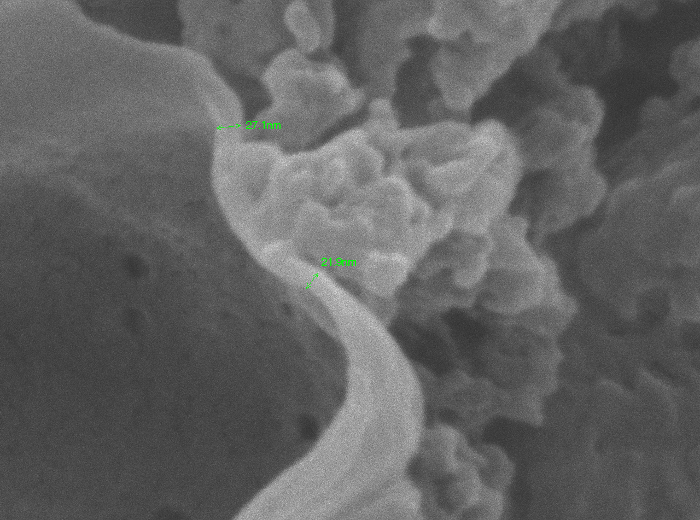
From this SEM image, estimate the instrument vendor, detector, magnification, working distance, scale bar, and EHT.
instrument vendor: JEOL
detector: SEI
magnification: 150 K X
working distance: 9.9 mm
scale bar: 100 nm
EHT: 10 kV